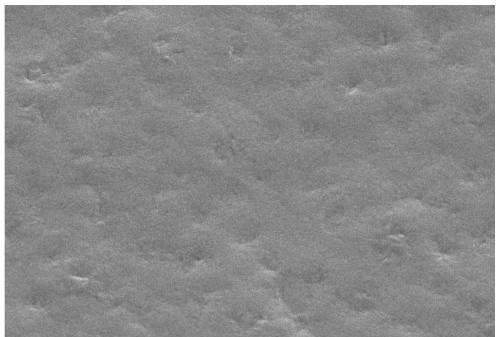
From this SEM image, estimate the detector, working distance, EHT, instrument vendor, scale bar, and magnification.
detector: SED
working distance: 10 mm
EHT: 14 kV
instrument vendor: JEOL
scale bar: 10000 nm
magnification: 2 K X